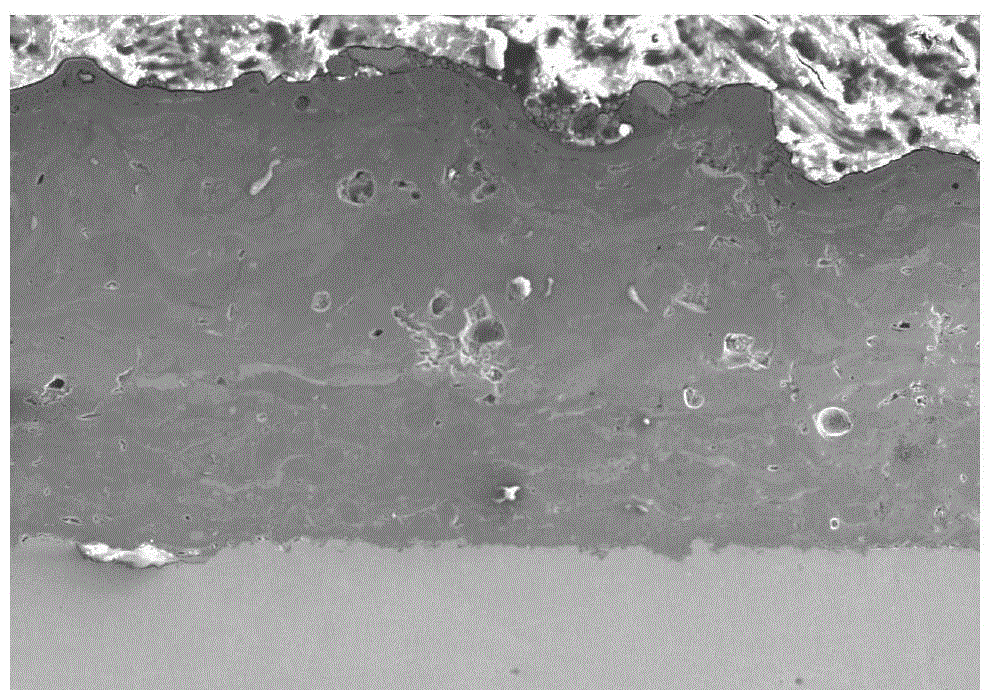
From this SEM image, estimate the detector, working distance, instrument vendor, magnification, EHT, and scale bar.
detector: SE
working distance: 10.3 mm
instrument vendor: Hitachi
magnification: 0.1 K X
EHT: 15 kV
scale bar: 500000 nm